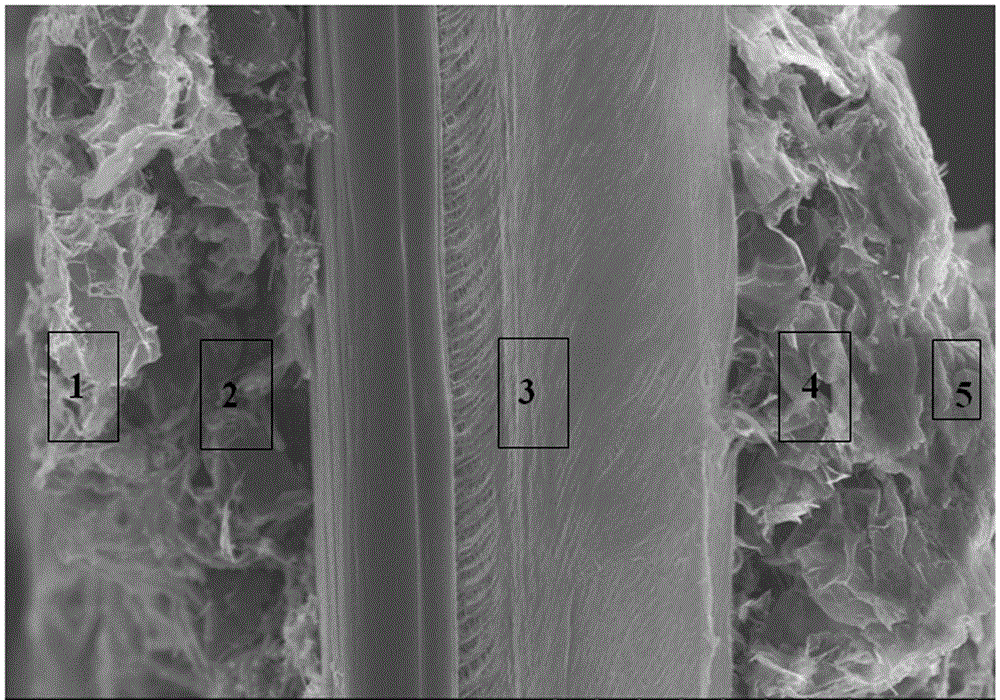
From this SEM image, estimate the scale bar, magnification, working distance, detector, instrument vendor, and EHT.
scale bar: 30000 nm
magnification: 1.8 K X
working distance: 8.2 mm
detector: SE(M)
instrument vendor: Hitachi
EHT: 10 kV